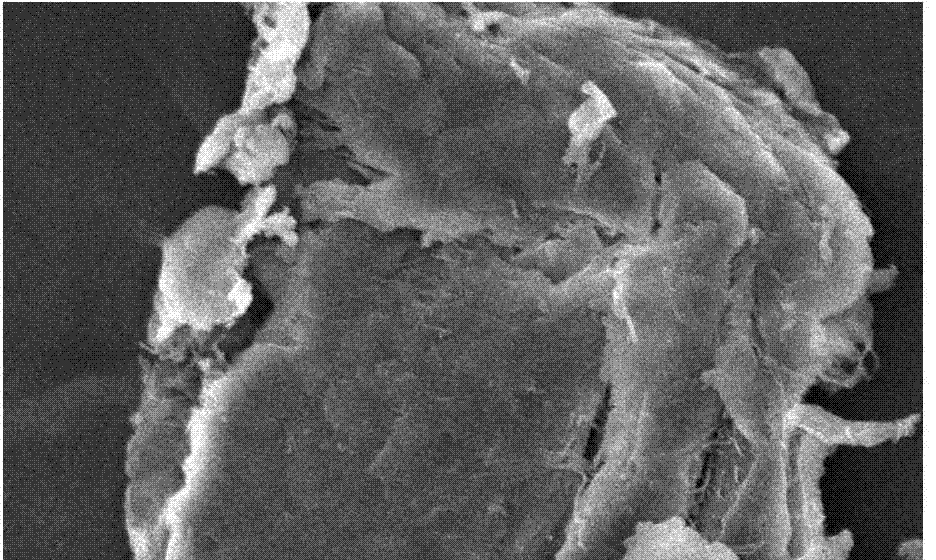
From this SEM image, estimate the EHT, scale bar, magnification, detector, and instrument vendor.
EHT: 20 kV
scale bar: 10000 nm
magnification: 1 K X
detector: SEI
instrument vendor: JEOL